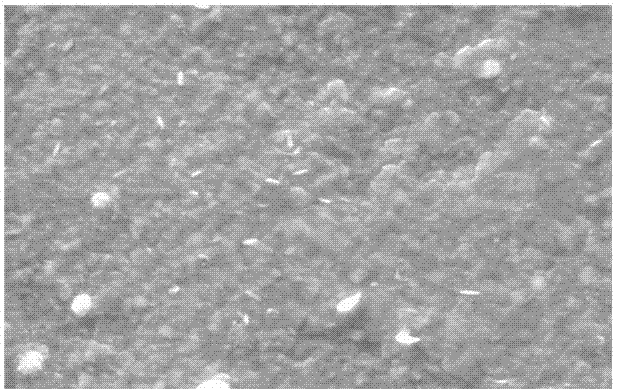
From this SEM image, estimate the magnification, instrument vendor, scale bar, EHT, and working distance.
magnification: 25 K X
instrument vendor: Zeiss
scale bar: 1000 nm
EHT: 15 kV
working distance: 11.5 mm